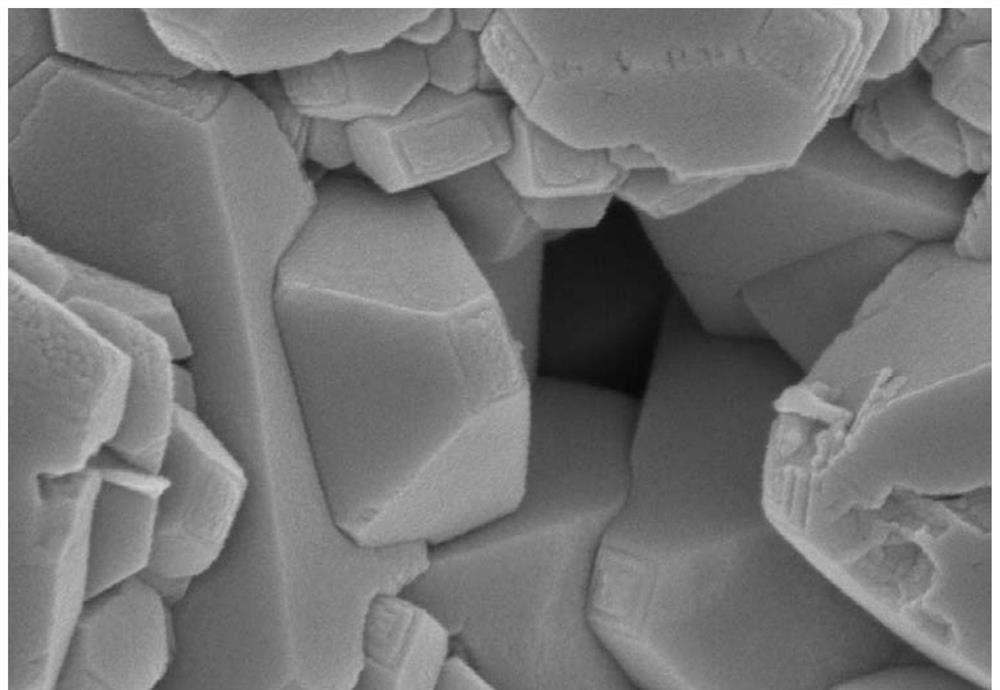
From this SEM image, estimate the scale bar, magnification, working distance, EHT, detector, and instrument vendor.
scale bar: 500 nm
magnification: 80 K X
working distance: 6 mm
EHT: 5 kV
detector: SE(M)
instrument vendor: Hitachi